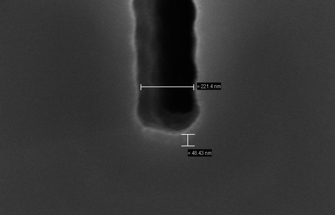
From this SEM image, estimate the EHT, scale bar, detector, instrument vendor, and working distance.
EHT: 5 kV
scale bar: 100 nm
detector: InLens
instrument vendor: Zeiss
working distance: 6.5 mm